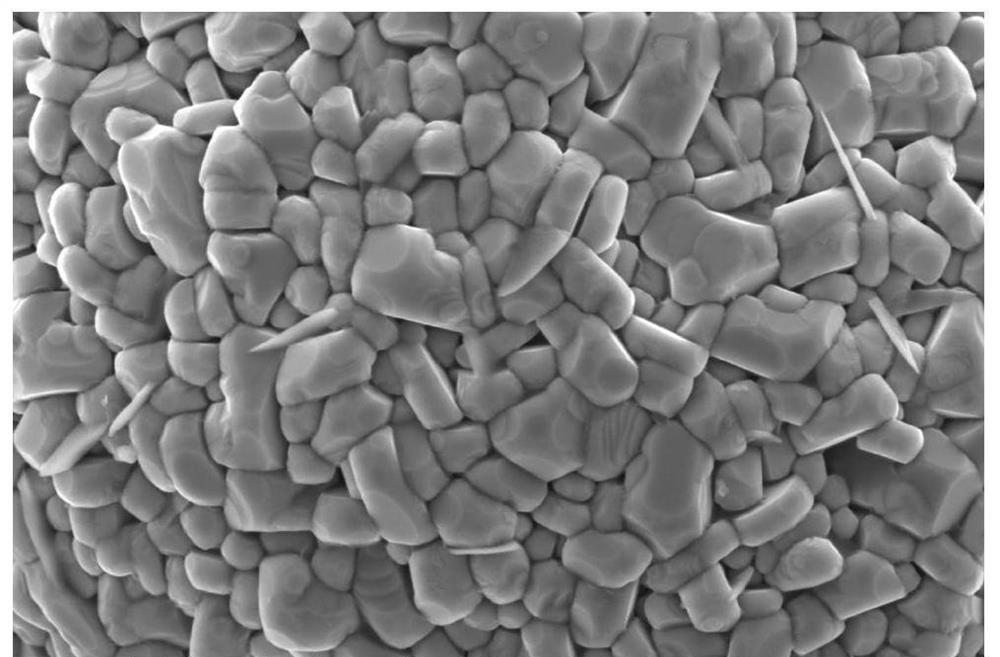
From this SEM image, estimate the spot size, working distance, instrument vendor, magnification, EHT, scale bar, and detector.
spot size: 3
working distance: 10.7 mm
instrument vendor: FEI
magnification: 50 K X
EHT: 5 kV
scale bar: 3000 nm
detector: ETD